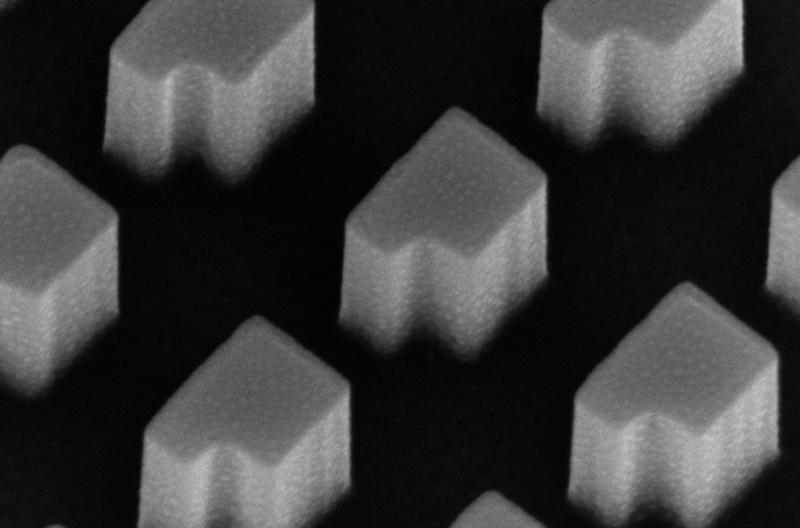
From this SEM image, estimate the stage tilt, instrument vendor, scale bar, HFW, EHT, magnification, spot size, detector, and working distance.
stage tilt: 30°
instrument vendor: FEI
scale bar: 500 nm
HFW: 1.95 µm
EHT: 10 kV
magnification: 65 K X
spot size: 3.5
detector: TLD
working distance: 4.4 mm